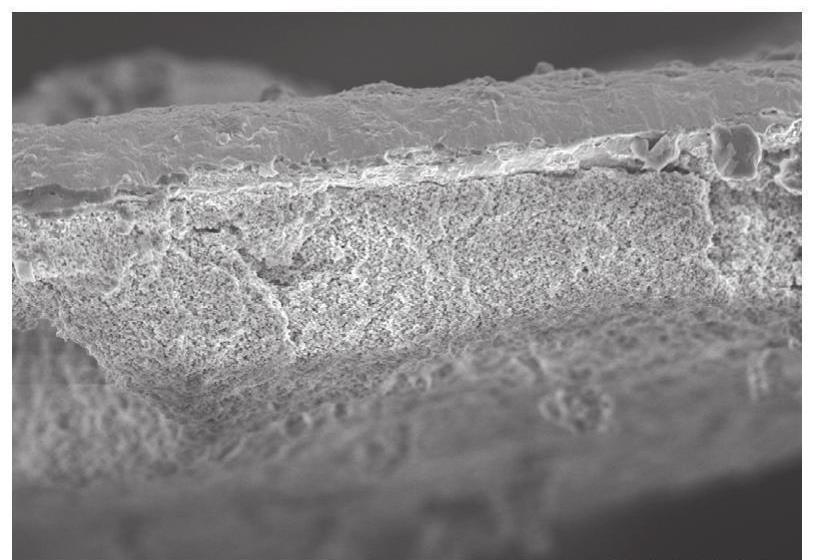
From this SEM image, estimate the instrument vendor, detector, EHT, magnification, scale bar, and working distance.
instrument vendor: Hitachi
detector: SE(U)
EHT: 5 kV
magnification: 3.5 K X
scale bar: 10000 nm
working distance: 8.3 mm